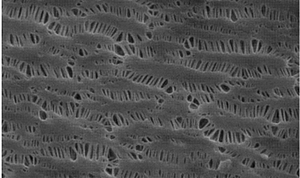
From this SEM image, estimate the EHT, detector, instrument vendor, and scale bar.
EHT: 15 kV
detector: SE1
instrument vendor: Zeiss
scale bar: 200 nm